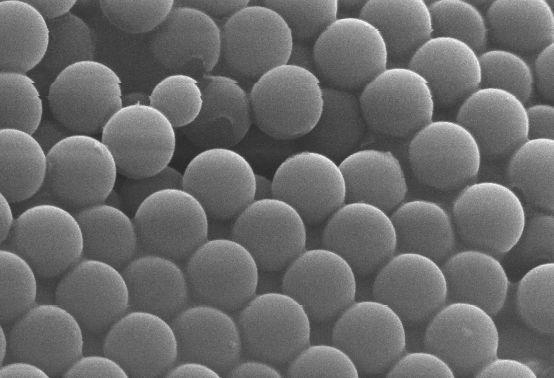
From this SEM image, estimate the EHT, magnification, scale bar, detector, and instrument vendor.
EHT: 30 kV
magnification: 7.5 K X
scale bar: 5000 nm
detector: SE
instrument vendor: other